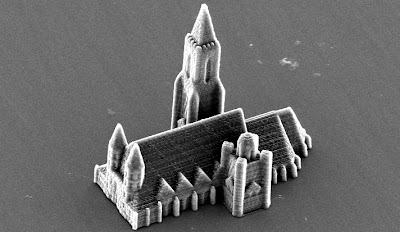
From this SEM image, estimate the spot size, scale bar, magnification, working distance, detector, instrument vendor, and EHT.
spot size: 4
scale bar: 20000 nm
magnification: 0.817 K X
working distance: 26.8 mm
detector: SE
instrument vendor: FEI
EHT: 12 kV